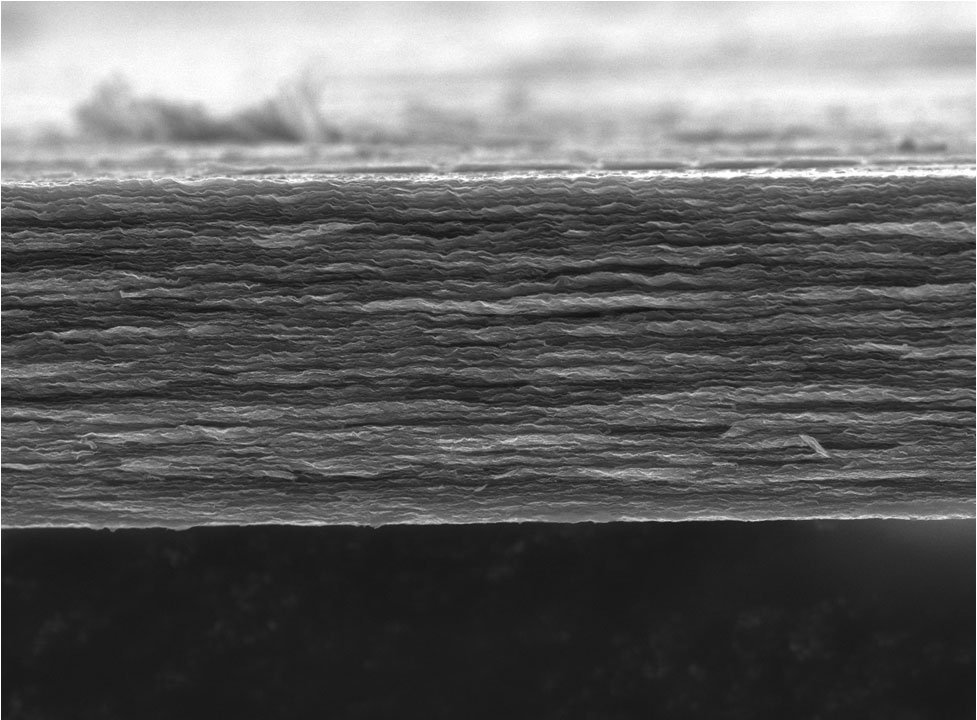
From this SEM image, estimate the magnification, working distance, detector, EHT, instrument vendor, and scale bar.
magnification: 5 K X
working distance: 9.9 mm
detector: SEI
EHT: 10 kV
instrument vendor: JEOL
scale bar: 1000 nm